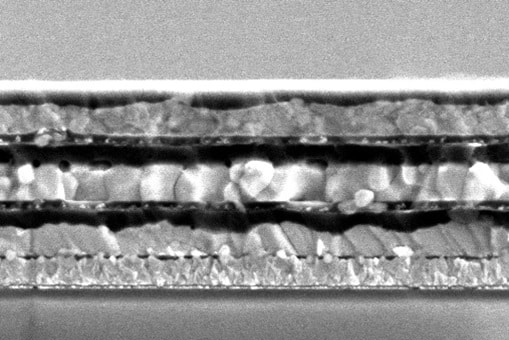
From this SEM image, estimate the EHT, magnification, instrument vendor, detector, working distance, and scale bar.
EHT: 3 kV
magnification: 20 K X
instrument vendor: JEOL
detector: SE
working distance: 10.5 mm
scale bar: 2000 nm